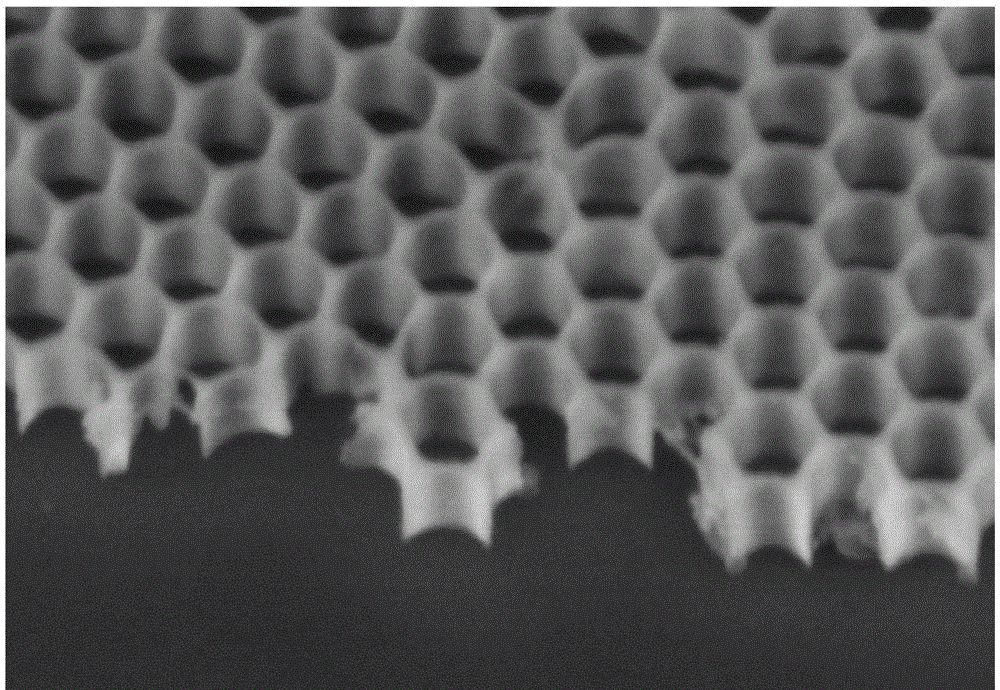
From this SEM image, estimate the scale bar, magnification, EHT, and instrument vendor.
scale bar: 400 nm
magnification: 120 K X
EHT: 3 kV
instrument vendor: Hitachi